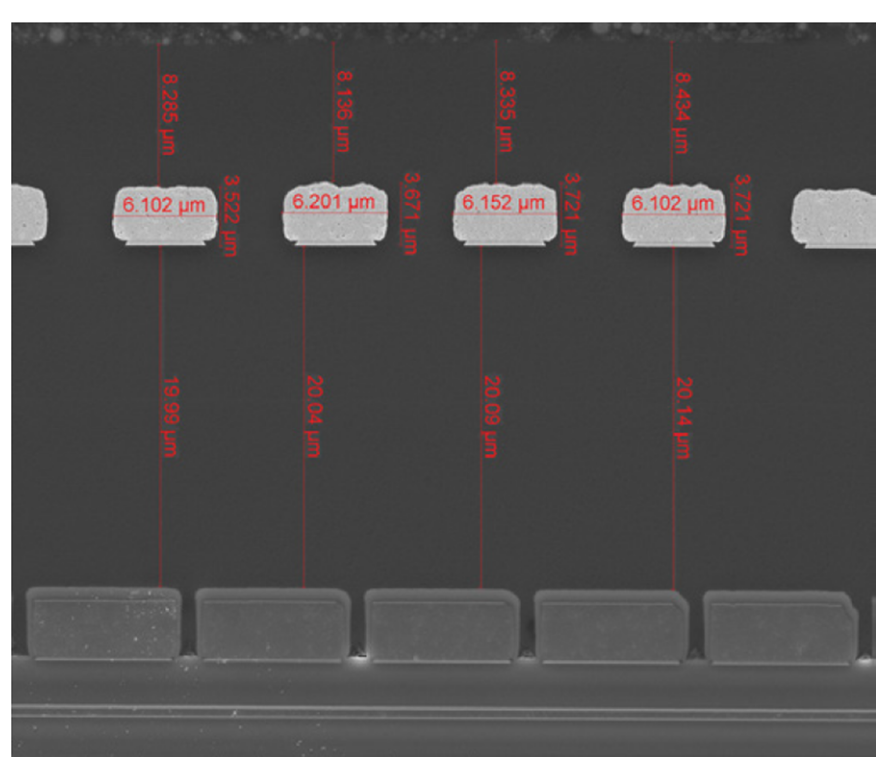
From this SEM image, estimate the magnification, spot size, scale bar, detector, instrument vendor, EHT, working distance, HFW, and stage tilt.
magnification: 2.5 K X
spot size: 3.5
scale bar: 20000 nm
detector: ETD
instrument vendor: FEI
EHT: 10 kV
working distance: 9 mm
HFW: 50.8 µm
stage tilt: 0°